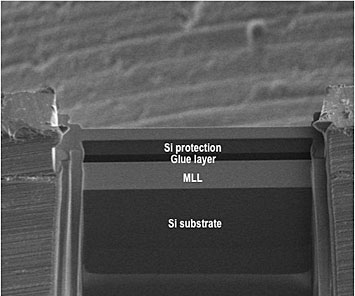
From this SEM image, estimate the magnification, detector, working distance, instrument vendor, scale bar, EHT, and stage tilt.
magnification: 0.5 K X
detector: ETD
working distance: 4 mm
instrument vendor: FEI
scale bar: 100000 nm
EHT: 5 kV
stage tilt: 53°